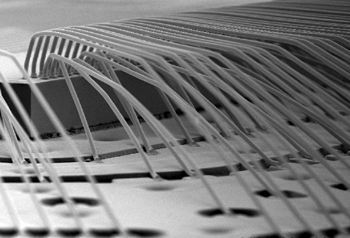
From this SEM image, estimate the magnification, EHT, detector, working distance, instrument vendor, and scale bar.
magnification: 0.06 K X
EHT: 15 kV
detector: SE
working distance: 26.5 mm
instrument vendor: Hitachi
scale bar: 500000 nm